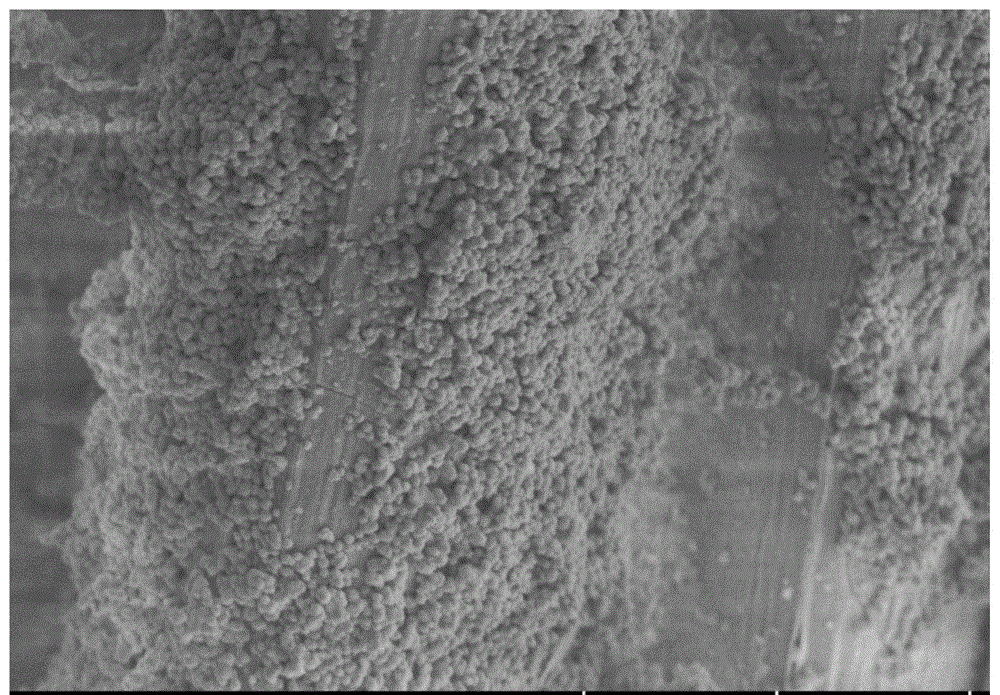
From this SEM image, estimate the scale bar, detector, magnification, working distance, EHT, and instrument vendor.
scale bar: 10000 nm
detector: SE(UL)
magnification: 5 K X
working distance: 10.6 mm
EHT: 3 kV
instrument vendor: Hitachi